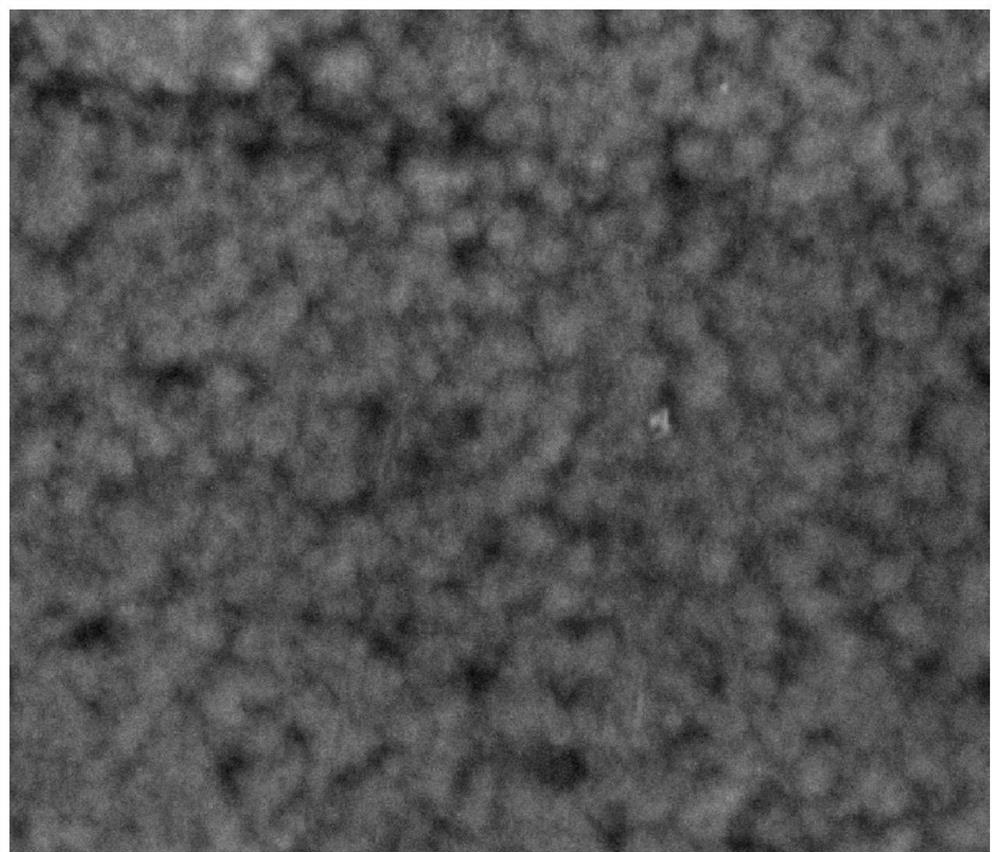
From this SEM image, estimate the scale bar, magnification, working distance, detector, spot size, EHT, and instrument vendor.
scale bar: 2000 nm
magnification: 50 K X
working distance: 4.9 mm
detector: ETD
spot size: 3.5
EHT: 10 kV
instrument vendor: FEI